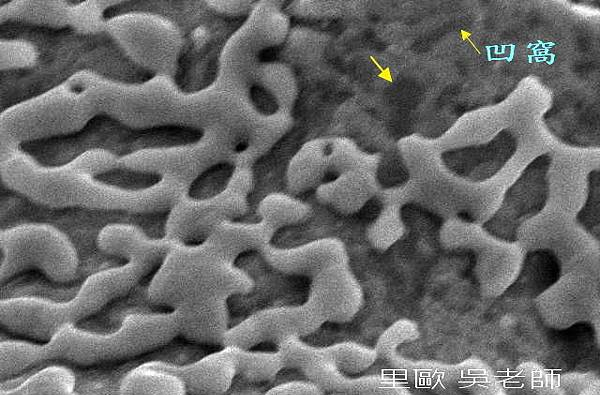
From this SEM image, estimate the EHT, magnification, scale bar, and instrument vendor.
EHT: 20 kV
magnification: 6 K X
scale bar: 2000 nm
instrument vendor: JEOL